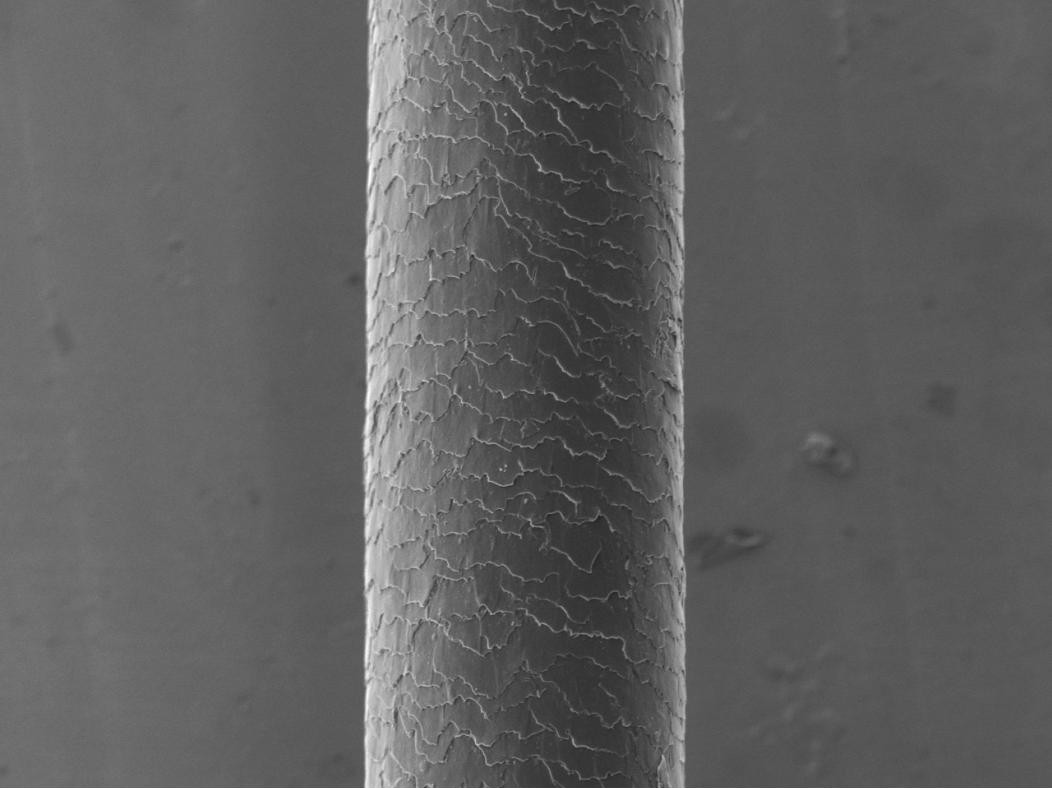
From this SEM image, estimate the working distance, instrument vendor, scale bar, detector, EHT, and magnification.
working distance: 9.6 mm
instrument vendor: JEOL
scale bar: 50000 nm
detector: SED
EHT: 5 kV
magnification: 0.5 K X